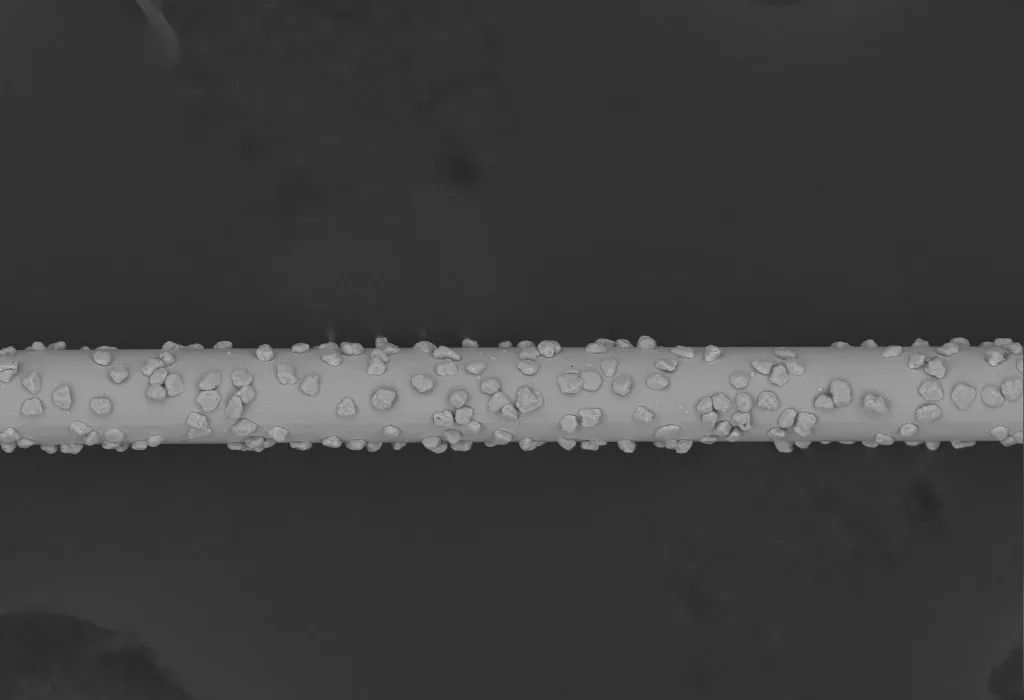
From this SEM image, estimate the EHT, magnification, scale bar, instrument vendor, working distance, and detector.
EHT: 20 kV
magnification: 0.25 K X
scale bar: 100000 nm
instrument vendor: other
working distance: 9 mm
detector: BSE